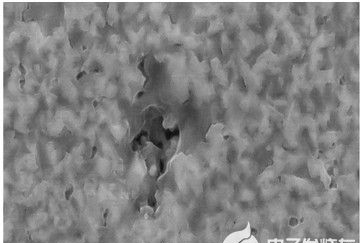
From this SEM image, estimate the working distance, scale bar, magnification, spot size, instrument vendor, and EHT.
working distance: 5 mm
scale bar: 1000 nm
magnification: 30 K X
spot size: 8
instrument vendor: other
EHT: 20 kV